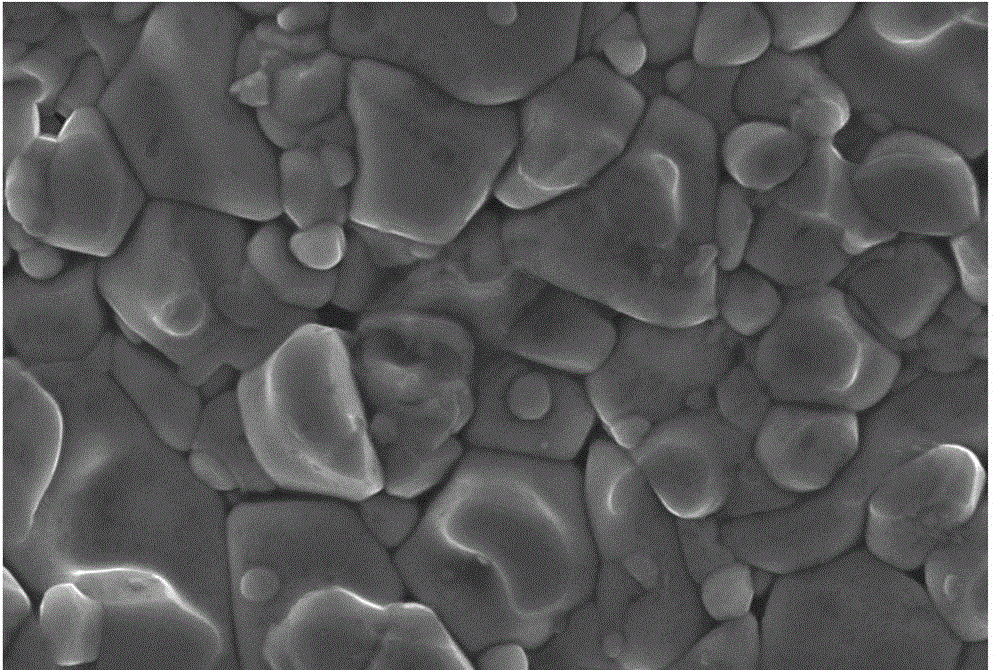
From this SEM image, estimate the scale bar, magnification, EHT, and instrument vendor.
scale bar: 5000 nm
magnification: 3 K X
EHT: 20 kV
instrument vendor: JEOL